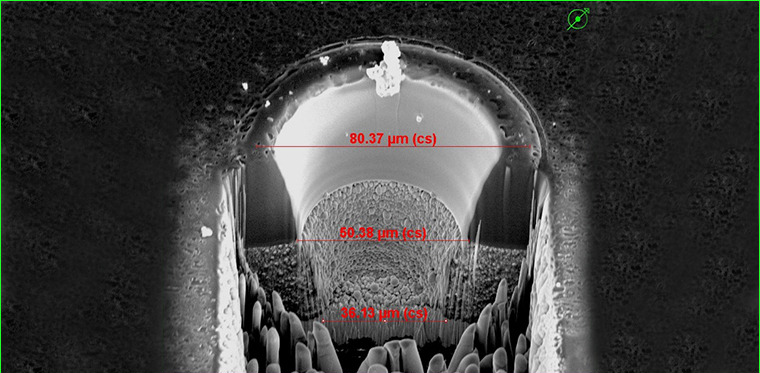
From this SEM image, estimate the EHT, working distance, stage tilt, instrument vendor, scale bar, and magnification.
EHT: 10 kV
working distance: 5.1 mm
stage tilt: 35°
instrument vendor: FEI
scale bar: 30000 nm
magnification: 1 K X